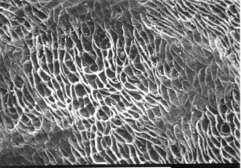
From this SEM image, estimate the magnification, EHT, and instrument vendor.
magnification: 0.5 K X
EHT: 20 kV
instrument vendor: JEOL